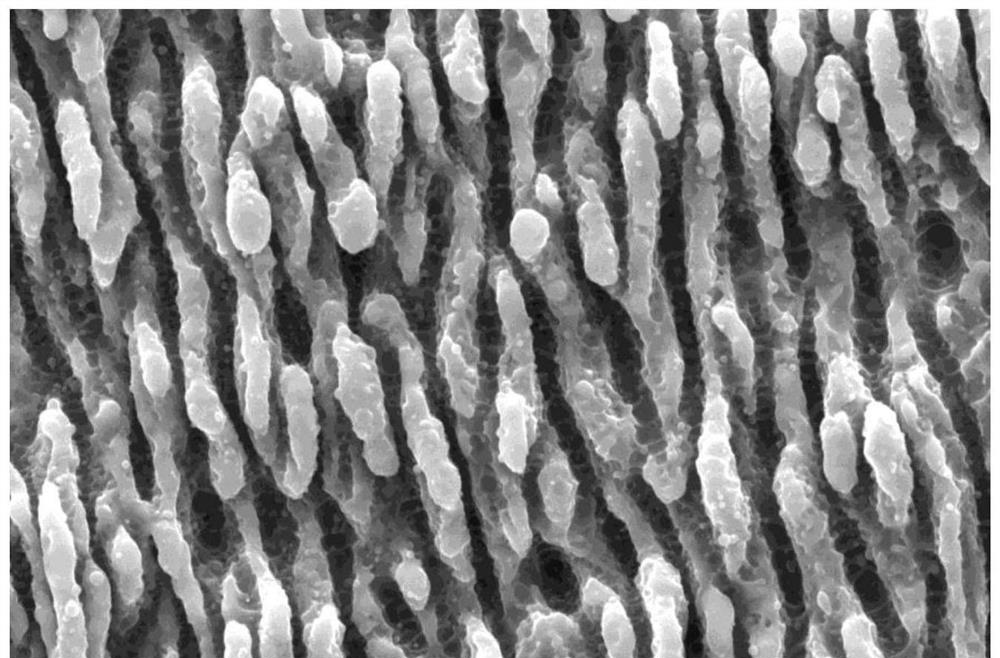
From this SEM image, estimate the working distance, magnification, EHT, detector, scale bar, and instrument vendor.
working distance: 10.3 mm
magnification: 40 K X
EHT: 10 kV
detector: ETD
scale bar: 3000 nm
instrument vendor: FEI